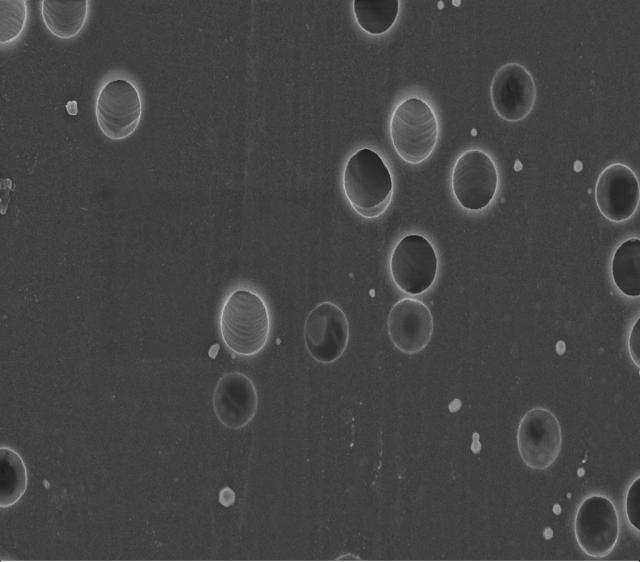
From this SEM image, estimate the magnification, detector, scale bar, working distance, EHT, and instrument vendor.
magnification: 30 K X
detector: InLens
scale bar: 1000 nm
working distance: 3 mm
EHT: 3 kV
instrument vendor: Zeiss